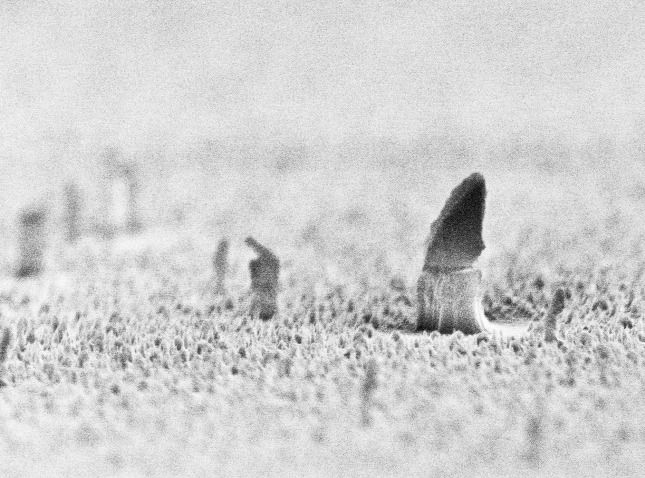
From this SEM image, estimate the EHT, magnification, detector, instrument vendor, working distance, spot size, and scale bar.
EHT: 10 kV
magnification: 24.008 K X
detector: TLD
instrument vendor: FEI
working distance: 5.6 mm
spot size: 2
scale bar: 1000 nm